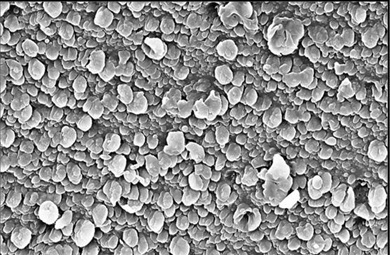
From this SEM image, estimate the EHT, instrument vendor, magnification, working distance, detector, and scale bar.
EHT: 20 kV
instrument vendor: Zeiss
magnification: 15.15 K X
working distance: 8.7 mm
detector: InLens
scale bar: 2000 nm